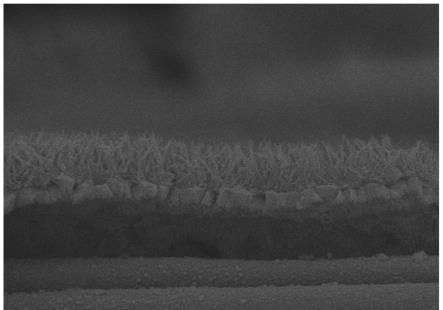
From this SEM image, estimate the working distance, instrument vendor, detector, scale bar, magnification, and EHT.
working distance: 6.6 mm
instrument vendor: Hitachi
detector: SE(M)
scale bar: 2000 nm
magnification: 20 K X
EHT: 5 kV